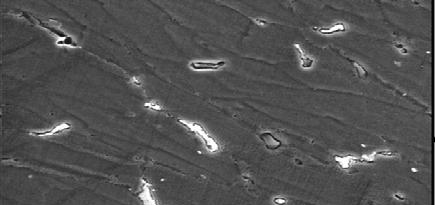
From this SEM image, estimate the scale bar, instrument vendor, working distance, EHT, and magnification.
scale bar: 10000 nm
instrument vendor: JEOL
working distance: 36 mm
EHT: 25 kV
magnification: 0.5 K X